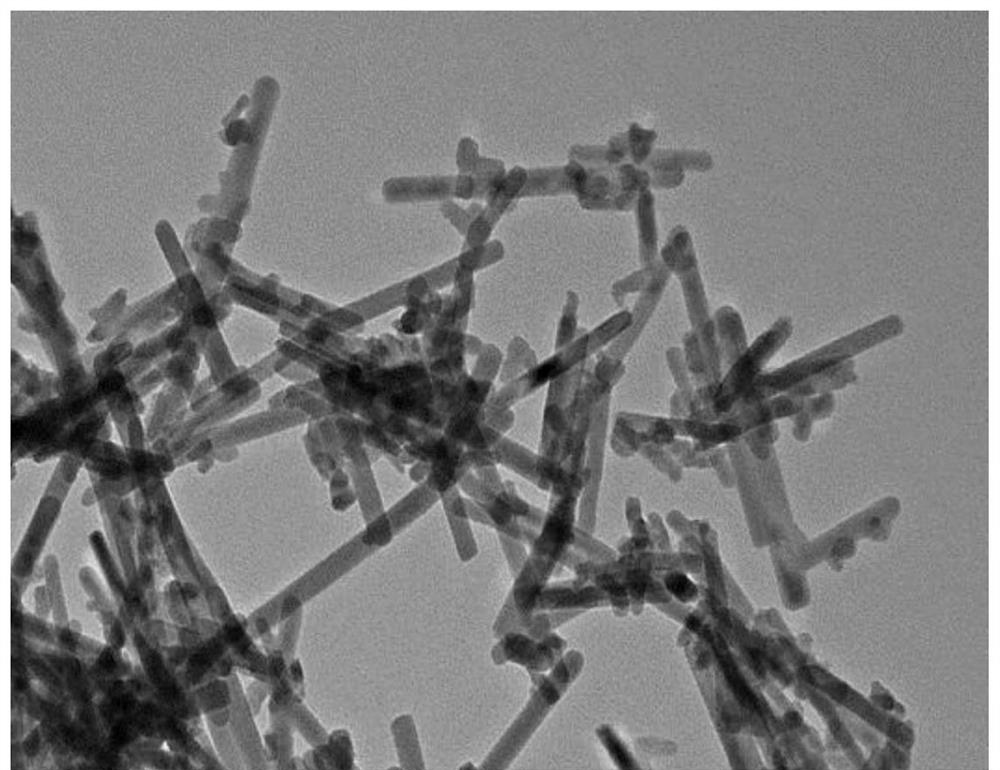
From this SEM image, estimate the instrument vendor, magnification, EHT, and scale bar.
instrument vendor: JEOL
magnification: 80 K X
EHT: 200 kV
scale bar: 100 nm